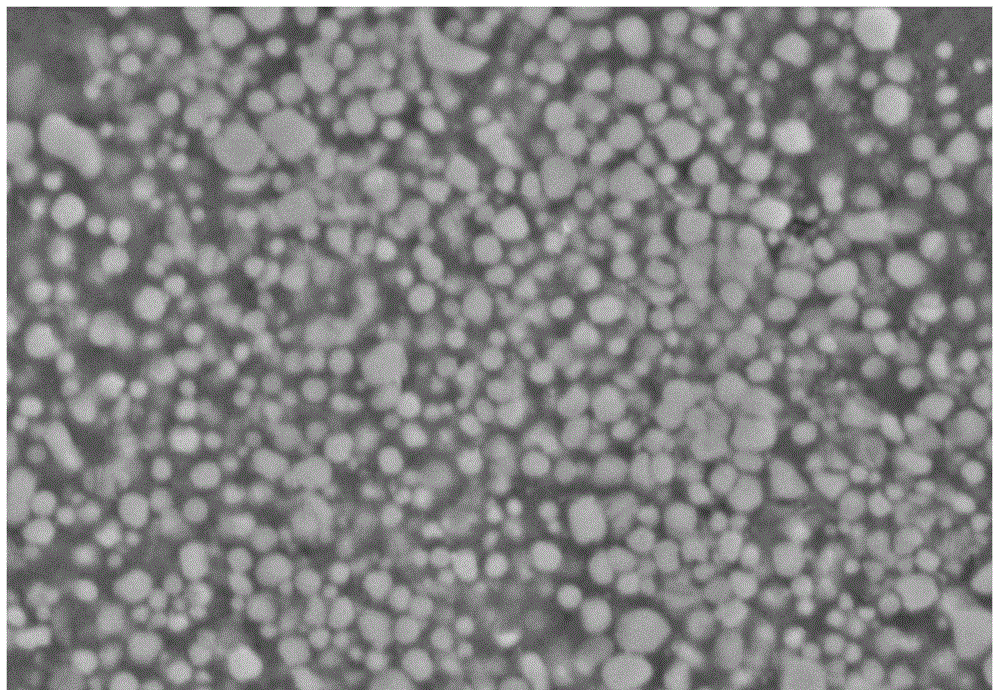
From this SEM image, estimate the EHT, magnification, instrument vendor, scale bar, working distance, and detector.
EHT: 15 kV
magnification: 5 K X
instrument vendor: Hitachi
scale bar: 10000 nm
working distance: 10 mm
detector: SE